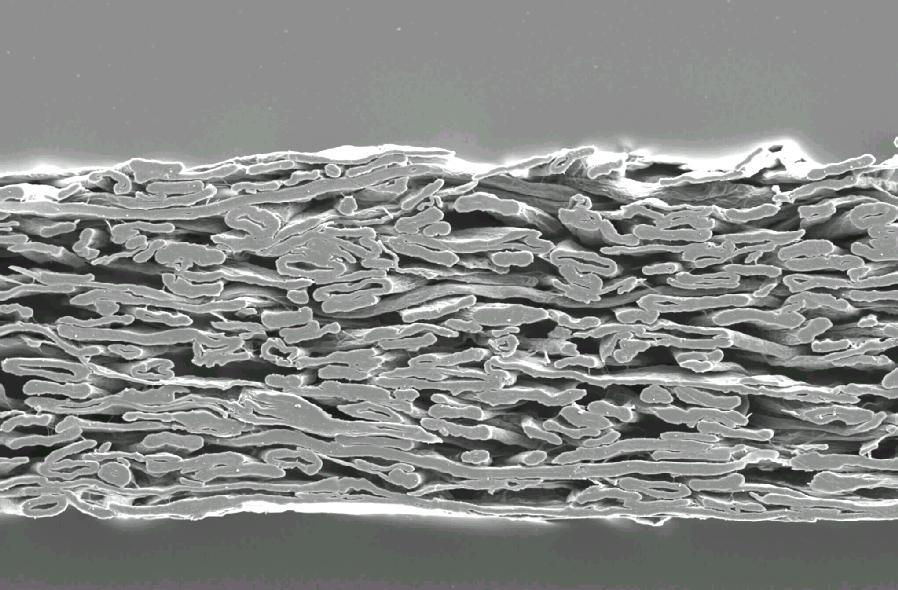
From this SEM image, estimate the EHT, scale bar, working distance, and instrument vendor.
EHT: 7 kV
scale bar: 10000 nm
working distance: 27 mm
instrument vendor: JEOL